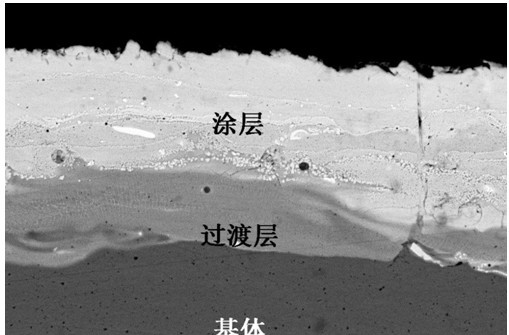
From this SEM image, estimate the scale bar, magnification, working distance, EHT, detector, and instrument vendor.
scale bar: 10000 nm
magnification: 2.5 K X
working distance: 20 mm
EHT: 20 kV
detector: QBSD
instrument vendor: Zeiss